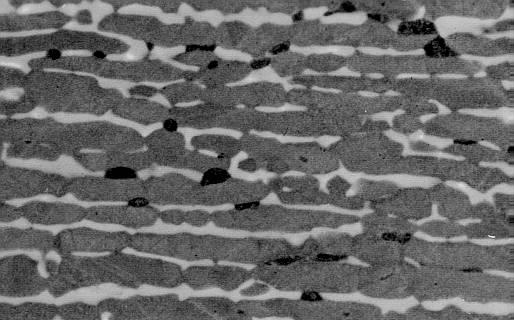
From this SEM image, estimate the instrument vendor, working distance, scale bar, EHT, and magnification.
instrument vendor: JEOL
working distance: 14 mm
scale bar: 10000 nm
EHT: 20 kV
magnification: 2.5 K X